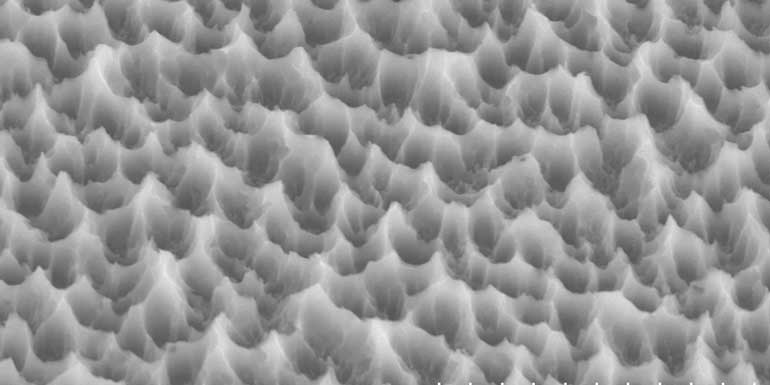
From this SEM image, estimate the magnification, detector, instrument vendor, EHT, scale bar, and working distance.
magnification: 13 K X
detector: SE(M)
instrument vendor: Hitachi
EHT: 7 kV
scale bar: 4000 nm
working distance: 19.4 mm